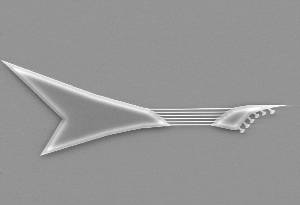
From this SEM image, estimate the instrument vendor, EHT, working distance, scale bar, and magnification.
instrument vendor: JEOL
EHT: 10 kV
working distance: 14 mm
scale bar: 10000 nm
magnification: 2.5 K X